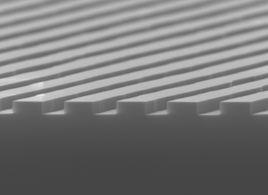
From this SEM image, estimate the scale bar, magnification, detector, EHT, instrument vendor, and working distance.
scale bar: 100 nm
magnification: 43 K X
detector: SEI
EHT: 8 kV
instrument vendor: JEOL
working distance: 7.5 mm